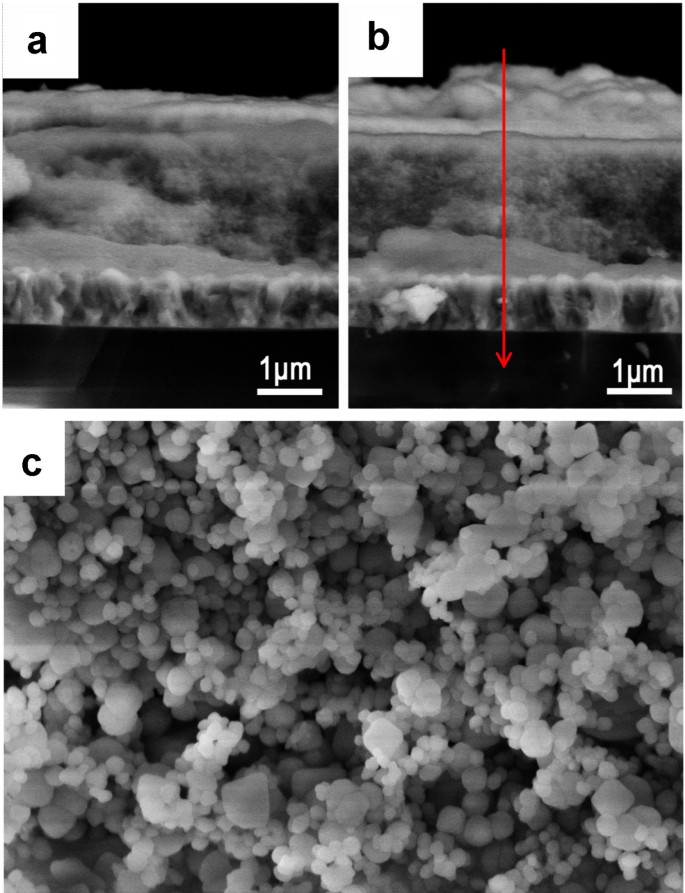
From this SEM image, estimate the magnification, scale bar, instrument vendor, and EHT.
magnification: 130 K X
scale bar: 400 nm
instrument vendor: Hitachi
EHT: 5 kV